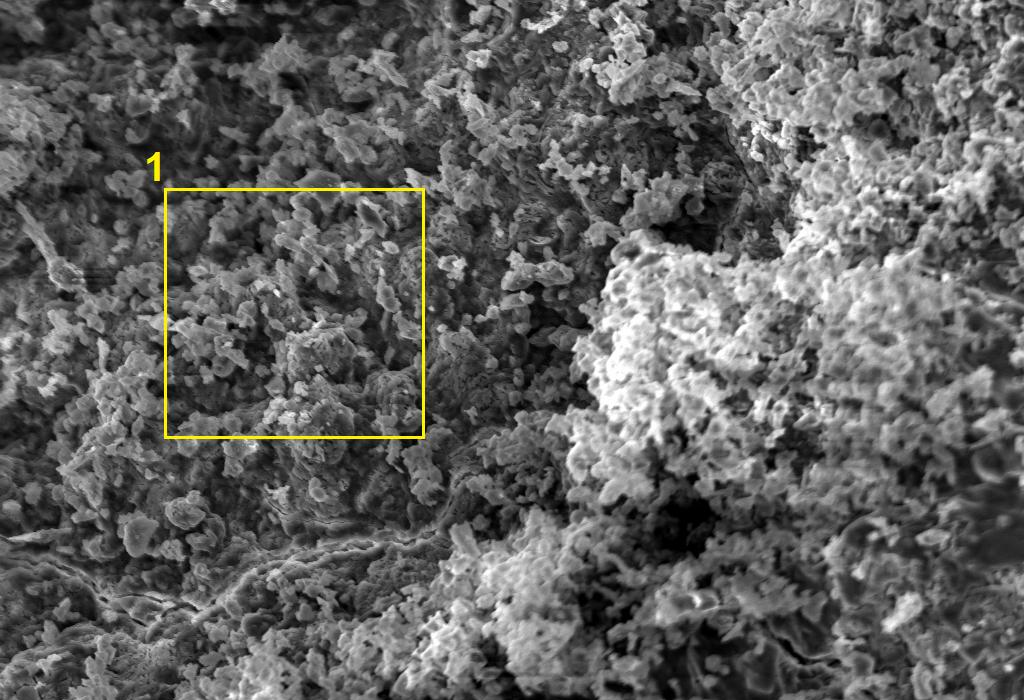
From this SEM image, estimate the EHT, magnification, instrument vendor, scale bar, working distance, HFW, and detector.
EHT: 15 kV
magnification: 1 K X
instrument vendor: Zeiss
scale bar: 10000 nm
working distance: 12 mm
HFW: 297.8 µm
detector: SE1